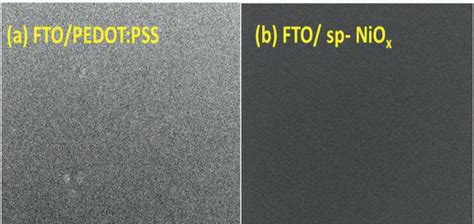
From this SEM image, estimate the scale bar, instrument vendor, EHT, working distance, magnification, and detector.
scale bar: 1000 nm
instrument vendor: JEOL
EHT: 5 kV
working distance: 9.2 mm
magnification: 19 K X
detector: SEI